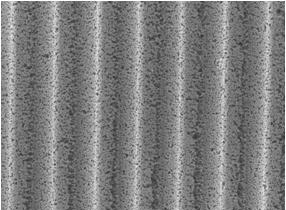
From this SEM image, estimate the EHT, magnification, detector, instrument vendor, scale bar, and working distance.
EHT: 10 kV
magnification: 0.8 K X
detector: LEI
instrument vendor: JEOL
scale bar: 10000 nm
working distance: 8 mm